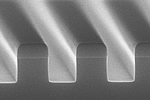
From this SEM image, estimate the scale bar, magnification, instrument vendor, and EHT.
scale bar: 1000 nm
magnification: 50 K X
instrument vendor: Hitachi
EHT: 10 kV